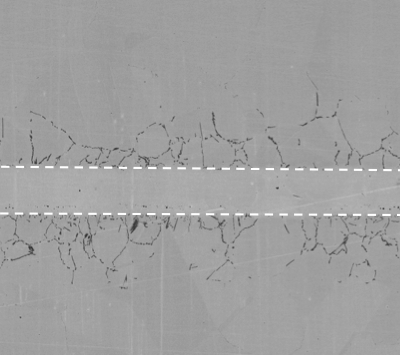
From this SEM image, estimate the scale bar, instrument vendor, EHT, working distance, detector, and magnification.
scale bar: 300000 nm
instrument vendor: Hitachi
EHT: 20 kV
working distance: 9.9 mm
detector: BSECOMP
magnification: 0.18 K X